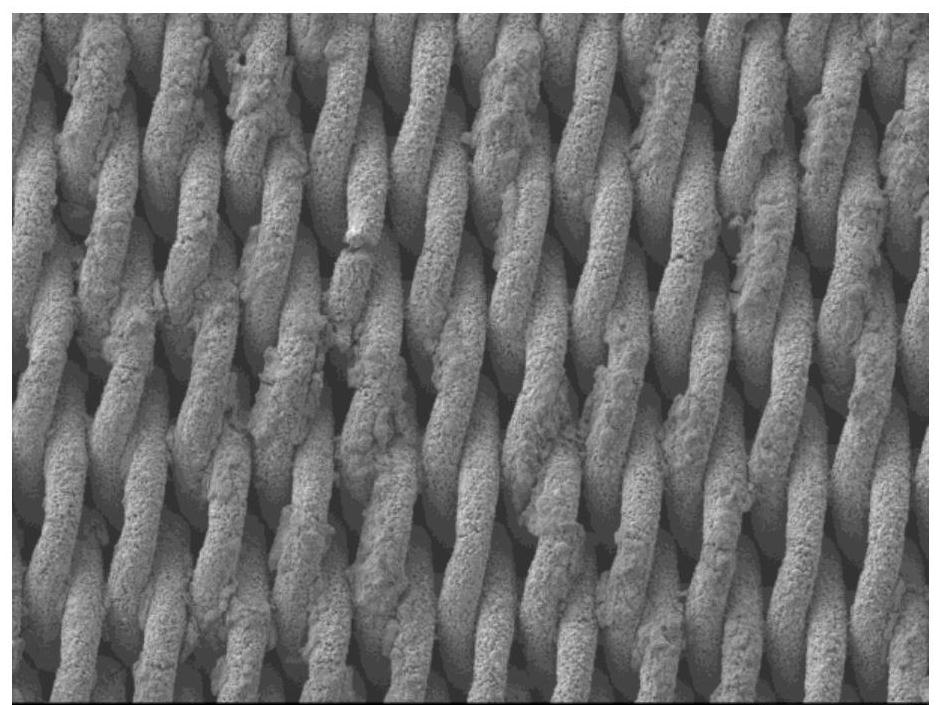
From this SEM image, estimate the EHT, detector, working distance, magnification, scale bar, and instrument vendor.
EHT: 5 kV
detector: LEI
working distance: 8 mm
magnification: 0.2 K X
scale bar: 100000 nm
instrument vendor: JEOL